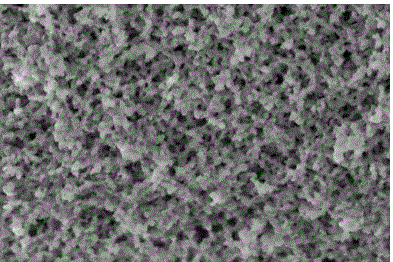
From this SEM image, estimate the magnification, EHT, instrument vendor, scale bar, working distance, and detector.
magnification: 500 K X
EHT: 1 kV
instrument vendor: FEI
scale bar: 300 nm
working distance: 2.7 mm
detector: TLD